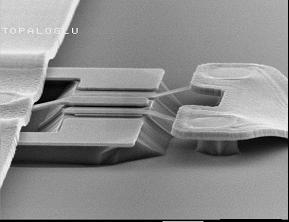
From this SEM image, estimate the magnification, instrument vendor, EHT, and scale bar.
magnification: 3.5 K X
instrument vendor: JEOL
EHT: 20 kV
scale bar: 5000 nm